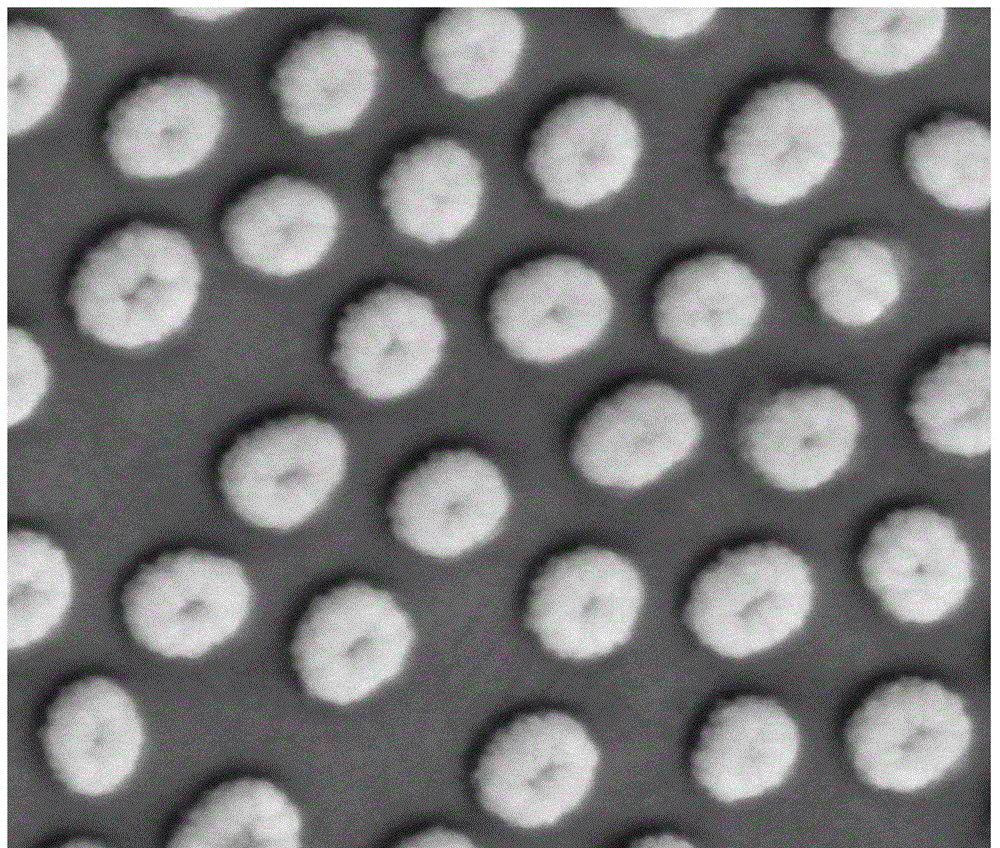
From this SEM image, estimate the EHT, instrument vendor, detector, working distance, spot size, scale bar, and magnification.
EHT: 20 kV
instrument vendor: FEI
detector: LFD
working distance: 10 mm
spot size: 3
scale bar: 1000 nm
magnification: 100 K X